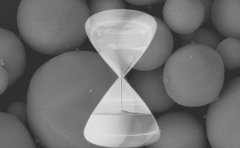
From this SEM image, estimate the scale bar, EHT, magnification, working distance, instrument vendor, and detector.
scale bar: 10000 nm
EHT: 20 kV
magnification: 5 K X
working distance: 10.1 mm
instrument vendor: Hitachi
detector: BSE3D